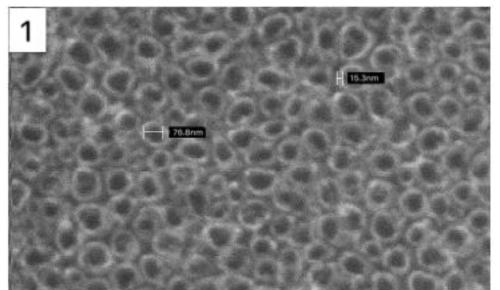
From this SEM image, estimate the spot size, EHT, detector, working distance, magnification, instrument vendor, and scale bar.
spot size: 3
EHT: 20 kV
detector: SE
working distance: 10.1 mm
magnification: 80 K X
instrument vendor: FEI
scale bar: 200 nm